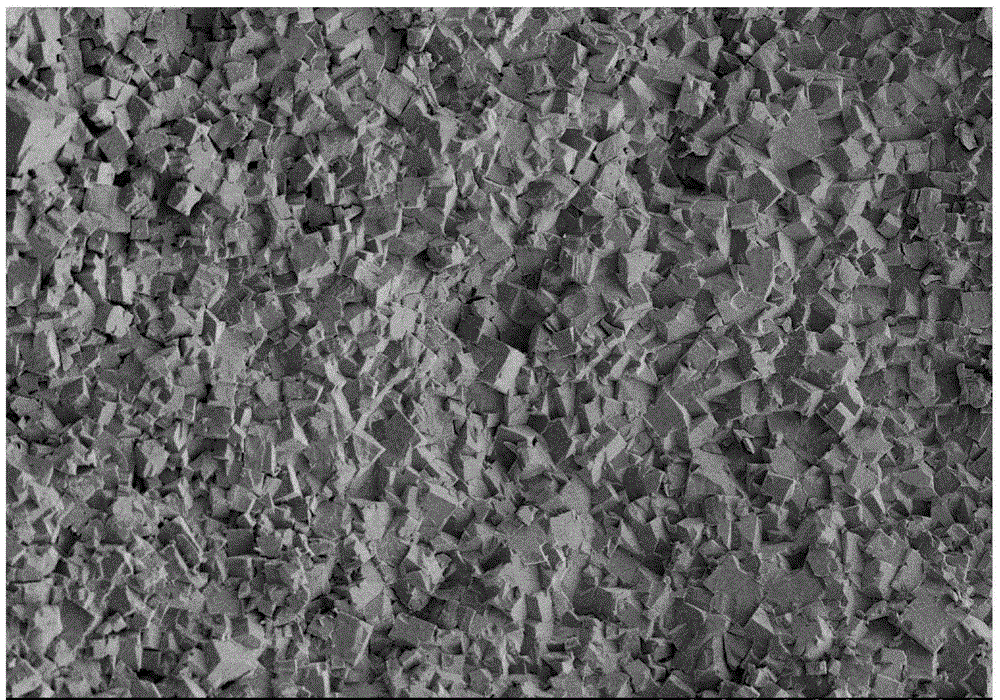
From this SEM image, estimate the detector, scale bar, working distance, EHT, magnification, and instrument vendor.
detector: SE(M,LA2)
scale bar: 20000 nm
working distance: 10.8 mm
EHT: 3 kV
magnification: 2 K X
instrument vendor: Hitachi